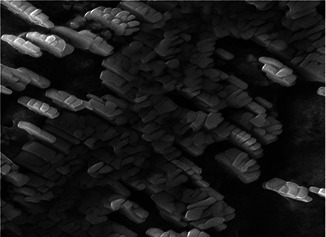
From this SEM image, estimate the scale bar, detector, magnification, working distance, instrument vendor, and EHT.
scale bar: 5000 nm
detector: SE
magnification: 10 K X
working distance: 15.19 mm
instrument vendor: Tescan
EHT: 20 kV